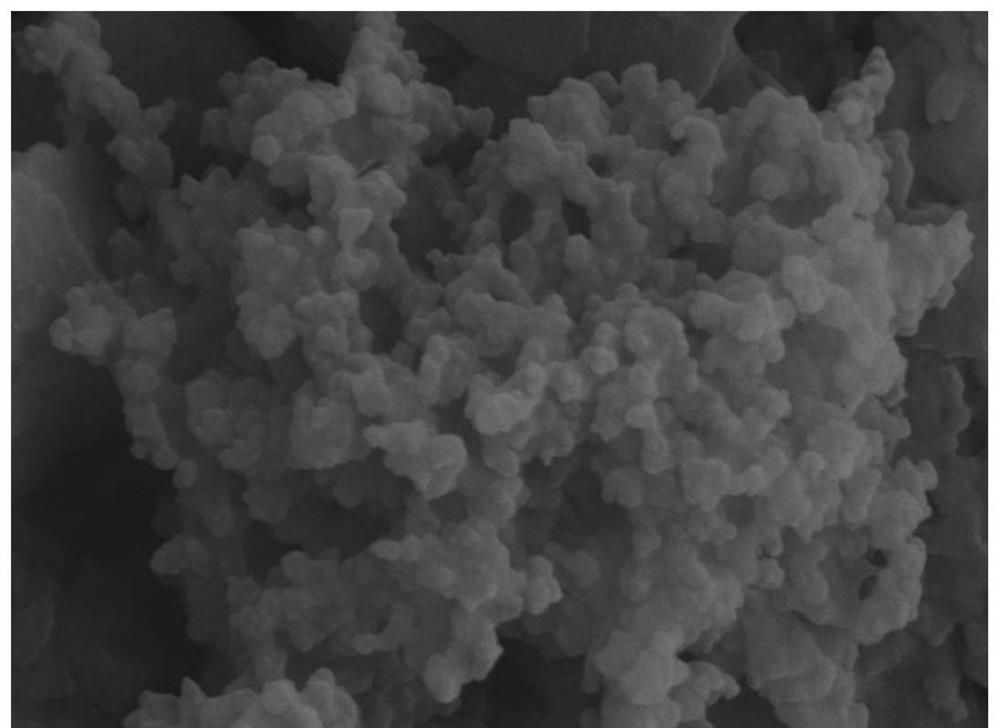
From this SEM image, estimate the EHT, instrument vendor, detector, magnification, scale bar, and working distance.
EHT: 5 kV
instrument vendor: Zeiss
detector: SE2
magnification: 50 K X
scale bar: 200 nm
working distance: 6.4 mm